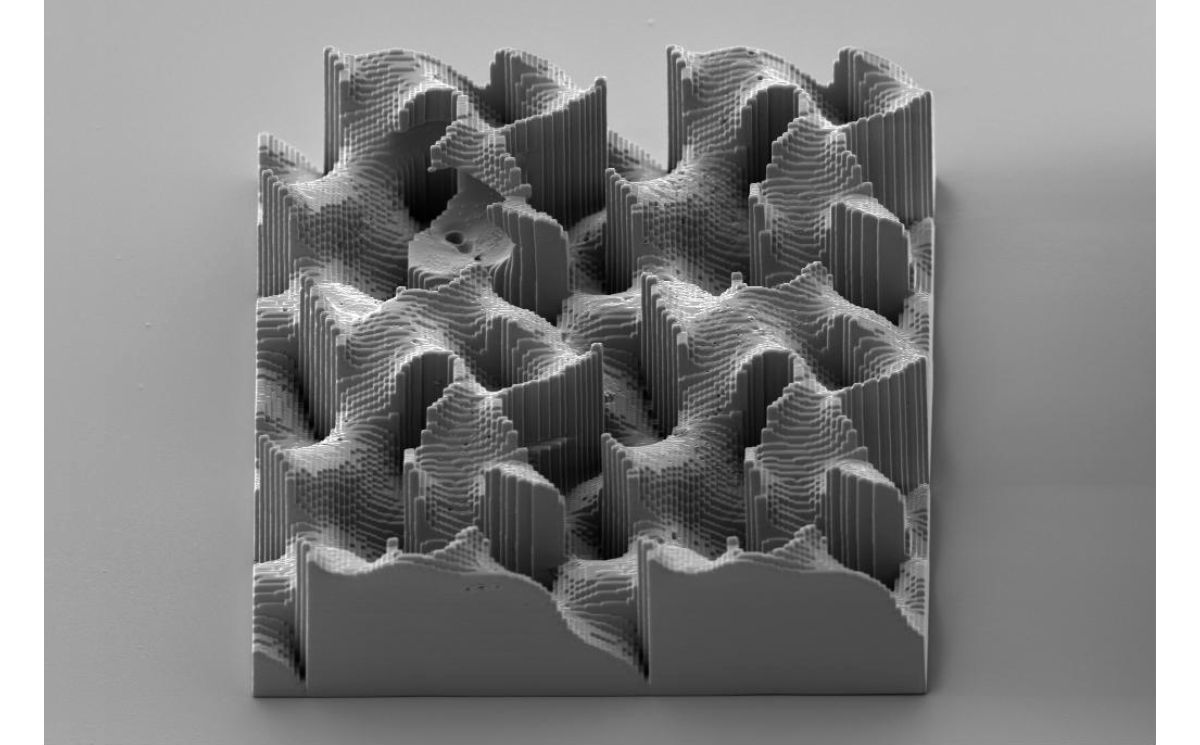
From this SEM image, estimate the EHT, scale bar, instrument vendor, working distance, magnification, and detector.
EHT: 2 kV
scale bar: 20000 nm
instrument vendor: Zeiss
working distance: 11.7 mm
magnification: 0.7 K X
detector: SE2